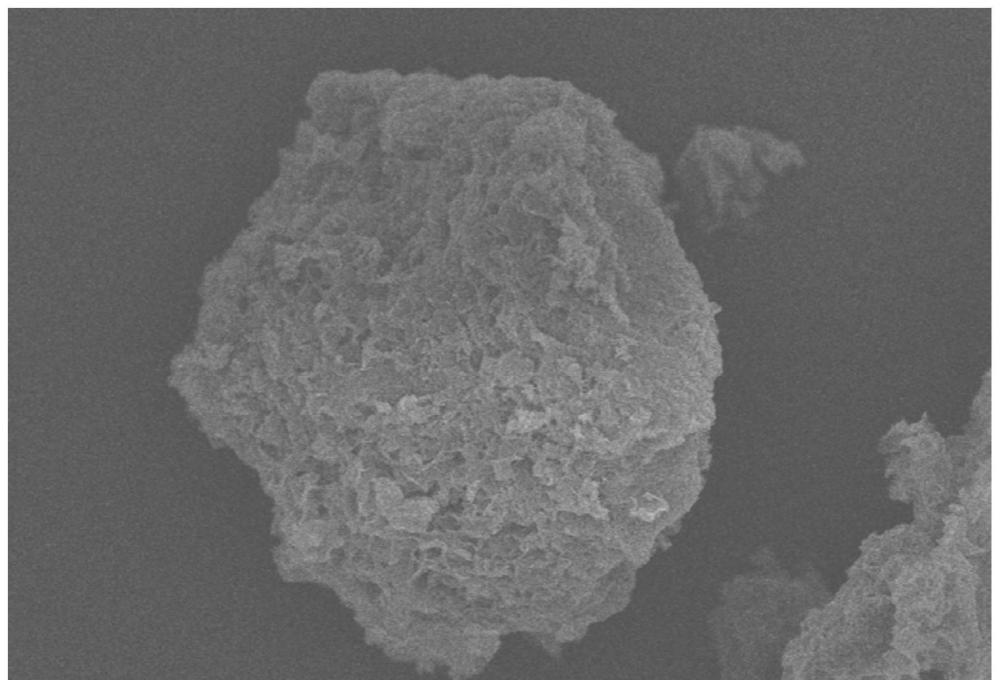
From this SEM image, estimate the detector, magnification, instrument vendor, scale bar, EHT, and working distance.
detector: SE(UL)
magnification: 5 K X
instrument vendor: Hitachi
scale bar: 10000 nm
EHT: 5 kV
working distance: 7.1 mm